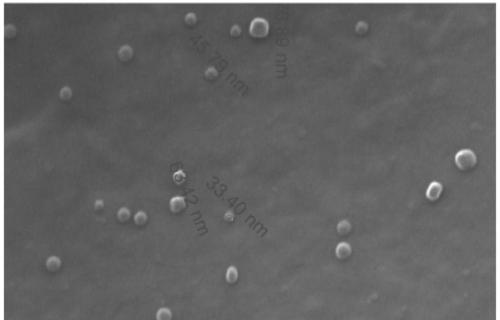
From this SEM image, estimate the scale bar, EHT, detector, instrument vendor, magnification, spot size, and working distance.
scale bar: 500 nm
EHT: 30 kV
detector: ETD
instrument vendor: FEI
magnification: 200 K X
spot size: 3.5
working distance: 7.4 mm